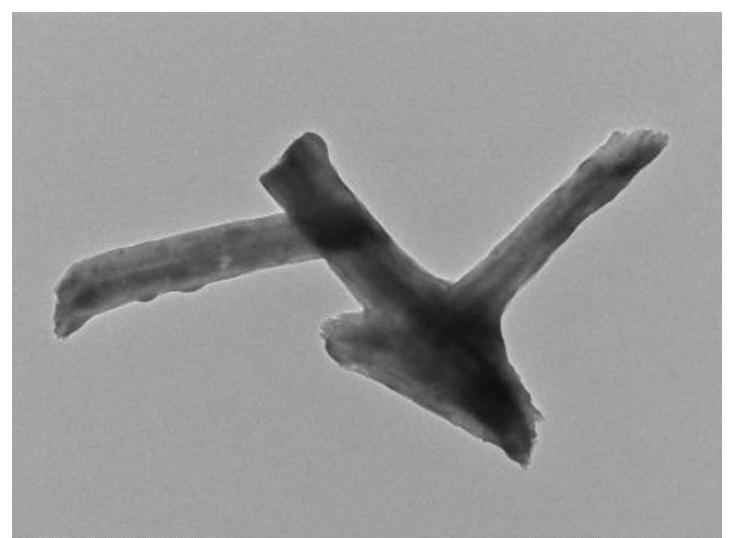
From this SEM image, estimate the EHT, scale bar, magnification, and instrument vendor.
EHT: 100 kV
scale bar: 1000 nm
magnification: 10 K X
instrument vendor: Hitachi